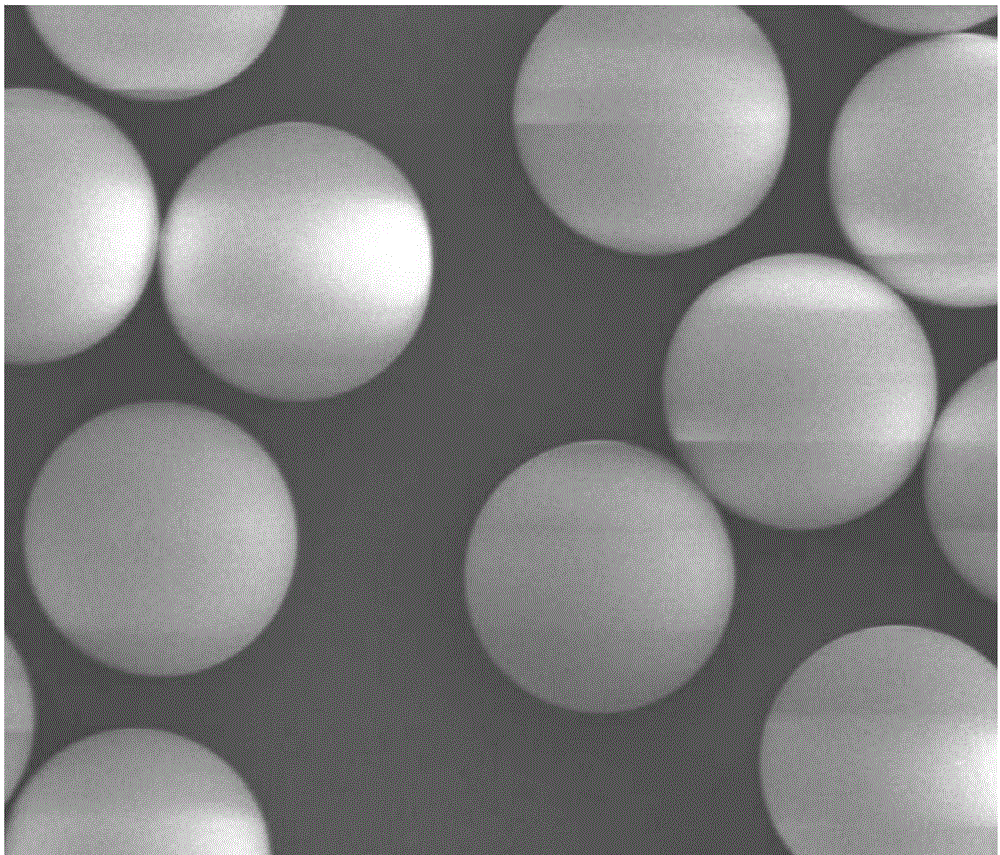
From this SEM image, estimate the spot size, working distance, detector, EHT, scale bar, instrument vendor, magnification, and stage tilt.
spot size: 3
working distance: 11.4 mm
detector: ETD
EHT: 5 kV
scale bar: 30000 nm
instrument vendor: FEI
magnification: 3 K X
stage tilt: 0°